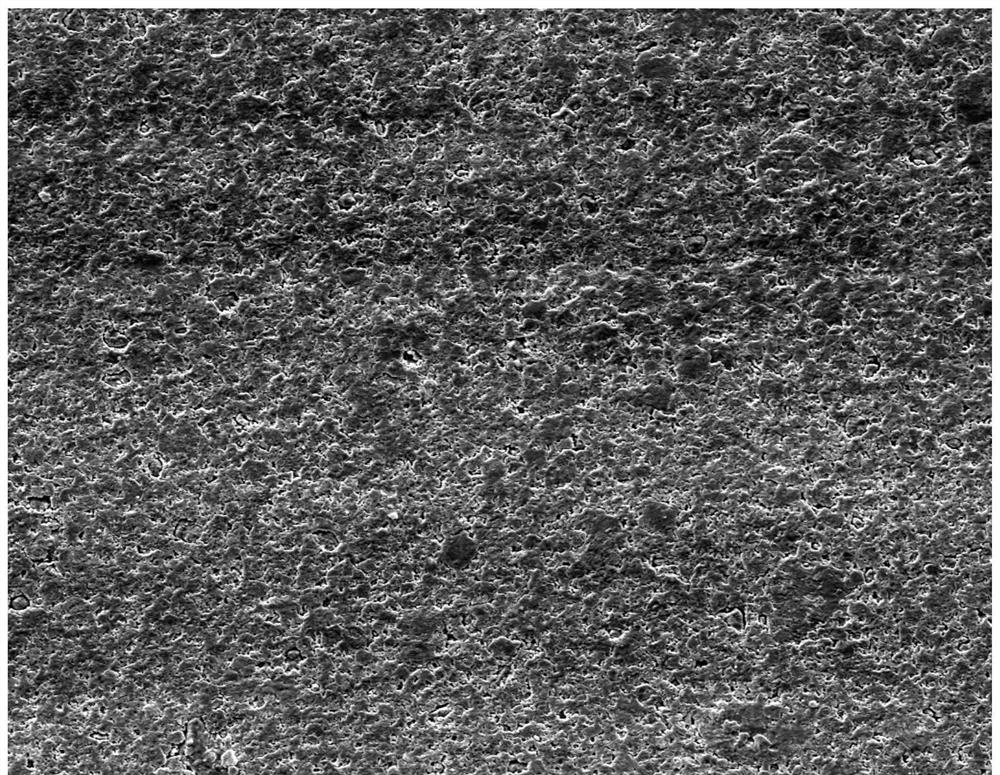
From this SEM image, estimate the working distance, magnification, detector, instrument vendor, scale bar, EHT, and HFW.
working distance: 10.4 mm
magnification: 0.5 K X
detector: ETD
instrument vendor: FEI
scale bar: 200000 nm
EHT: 30 kV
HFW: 597 µm